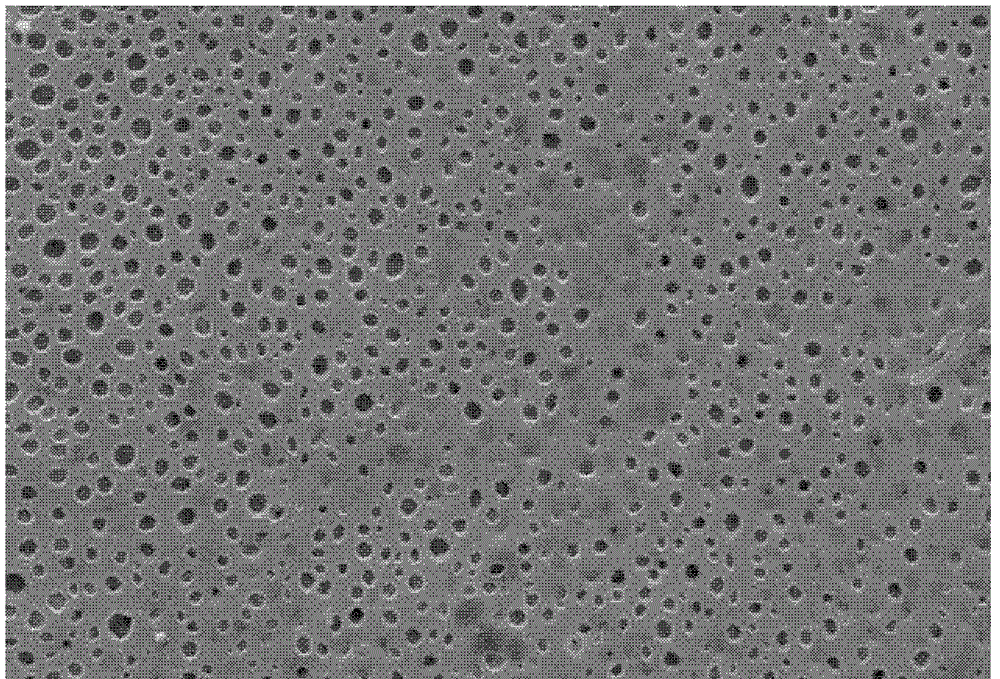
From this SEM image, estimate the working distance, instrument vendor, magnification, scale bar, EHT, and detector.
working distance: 20 mm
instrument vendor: Zeiss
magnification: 2 K X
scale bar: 3000 nm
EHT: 20 kV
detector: SE1